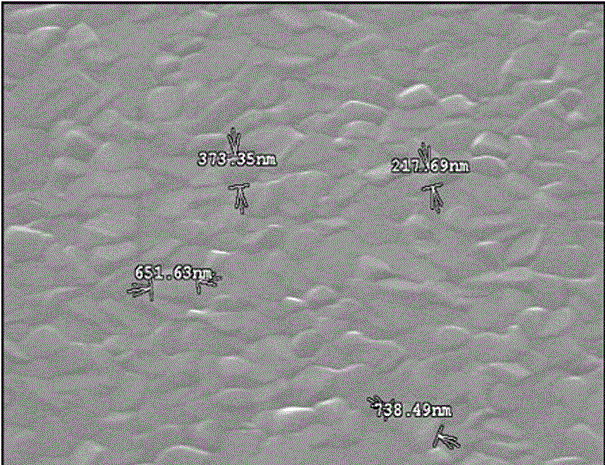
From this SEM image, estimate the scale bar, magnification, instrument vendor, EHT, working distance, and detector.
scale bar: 2000 nm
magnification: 15 K X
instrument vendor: Hitachi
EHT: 15 kV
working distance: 13.9 mm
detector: SE